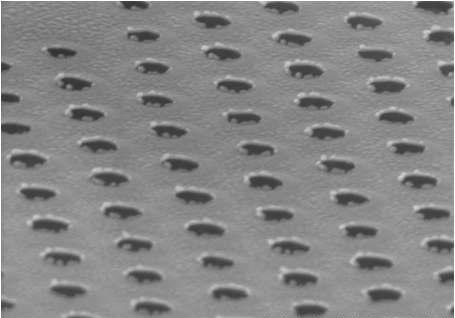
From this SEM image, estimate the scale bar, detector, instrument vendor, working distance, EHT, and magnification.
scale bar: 1000 nm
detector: SE(U)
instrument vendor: Hitachi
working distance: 13 mm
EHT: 10 kV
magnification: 50 K X